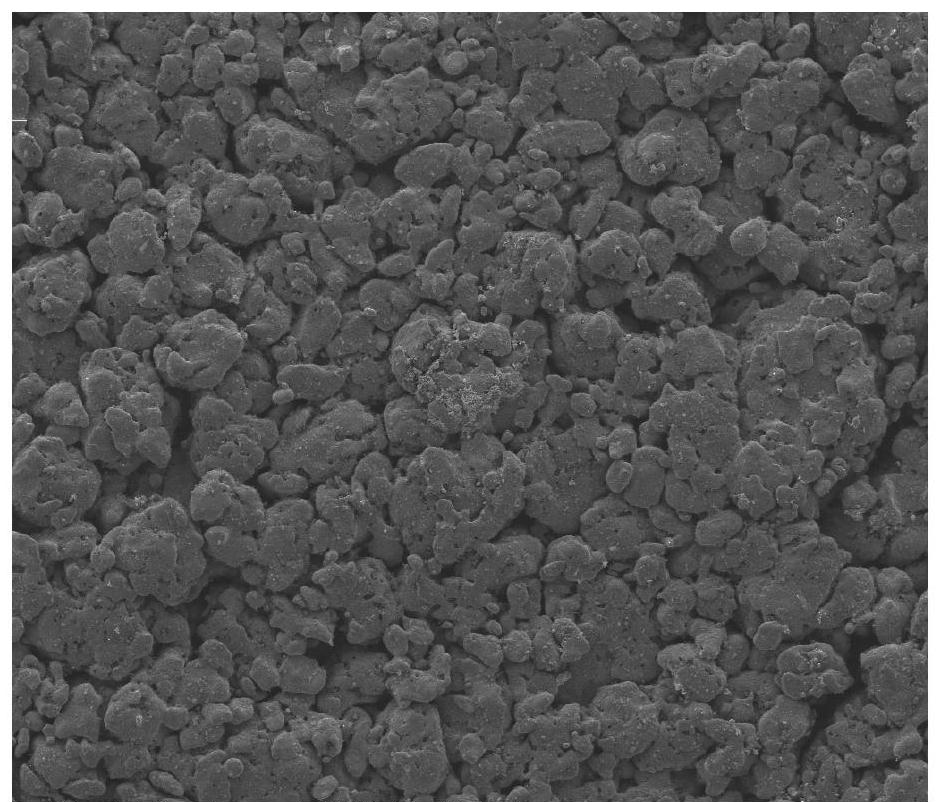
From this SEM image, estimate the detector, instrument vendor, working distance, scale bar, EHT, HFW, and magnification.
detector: ETD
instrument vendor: FEI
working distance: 10.7 mm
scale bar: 400000 nm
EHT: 20 kV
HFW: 995 µm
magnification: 0.3 K X